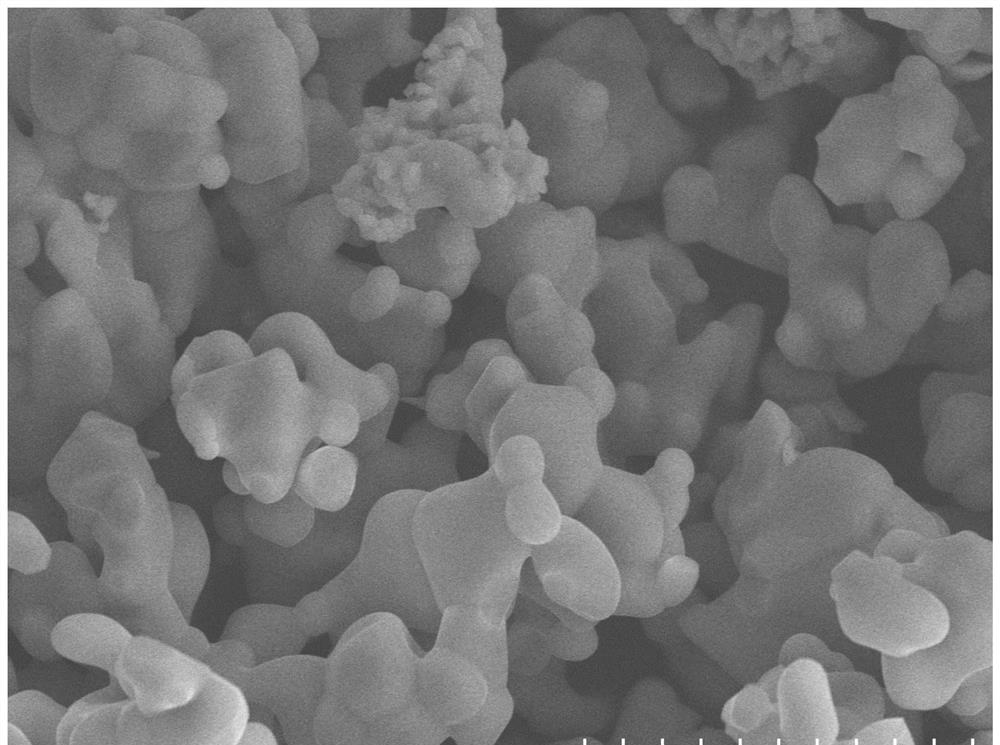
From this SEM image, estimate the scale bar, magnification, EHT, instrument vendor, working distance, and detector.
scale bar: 10000 nm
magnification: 5 K X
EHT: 15 kV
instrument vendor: Hitachi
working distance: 8.5 mm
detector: SE(M)